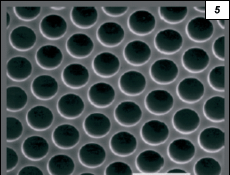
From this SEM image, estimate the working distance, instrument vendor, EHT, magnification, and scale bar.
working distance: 37 mm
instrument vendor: JEOL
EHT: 20 kV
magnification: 2.5 K X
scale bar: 10000 nm